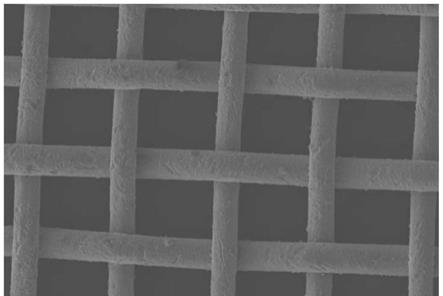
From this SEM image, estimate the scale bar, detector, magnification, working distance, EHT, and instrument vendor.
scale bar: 1000000 nm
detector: LM(UL)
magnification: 0.05 K X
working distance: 8 mm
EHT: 5 kV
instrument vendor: Hitachi